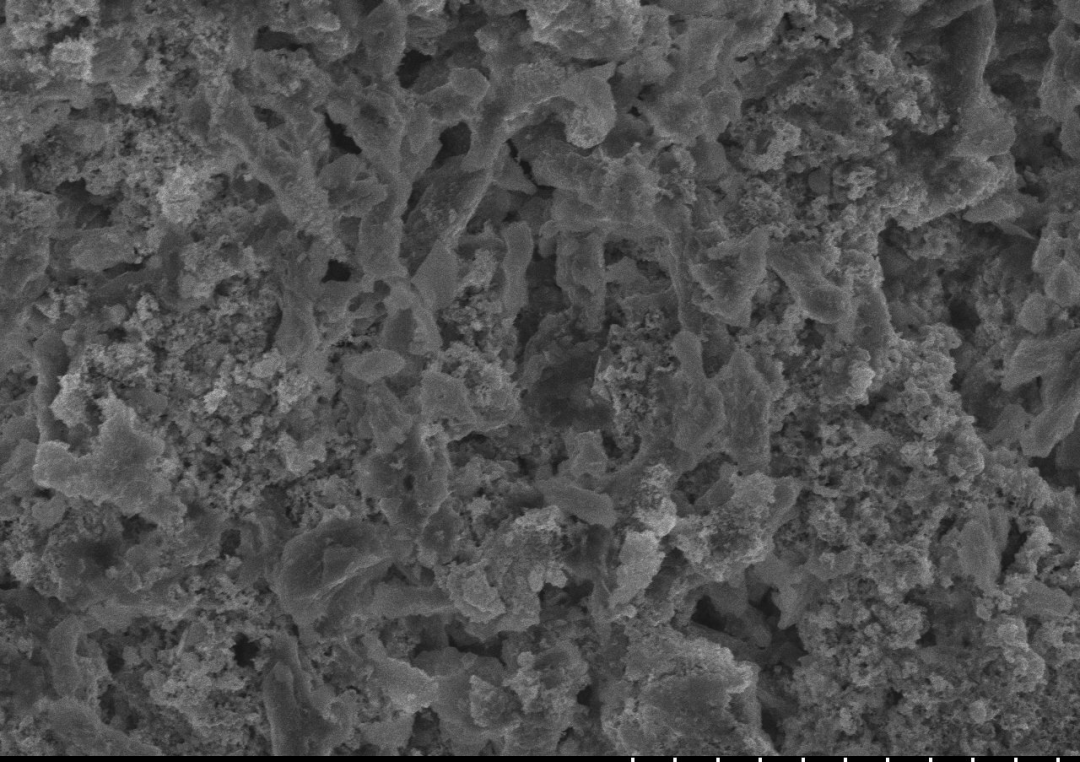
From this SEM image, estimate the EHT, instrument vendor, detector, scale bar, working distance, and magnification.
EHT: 20 kV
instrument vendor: Hitachi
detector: SE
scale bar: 50000 nm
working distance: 20.8 mm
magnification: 1 K X